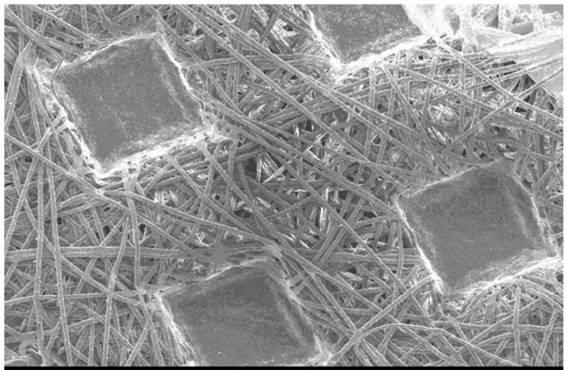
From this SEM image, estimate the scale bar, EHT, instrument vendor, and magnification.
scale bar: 500000 nm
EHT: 15 kV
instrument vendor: JEOL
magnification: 0.035 K X